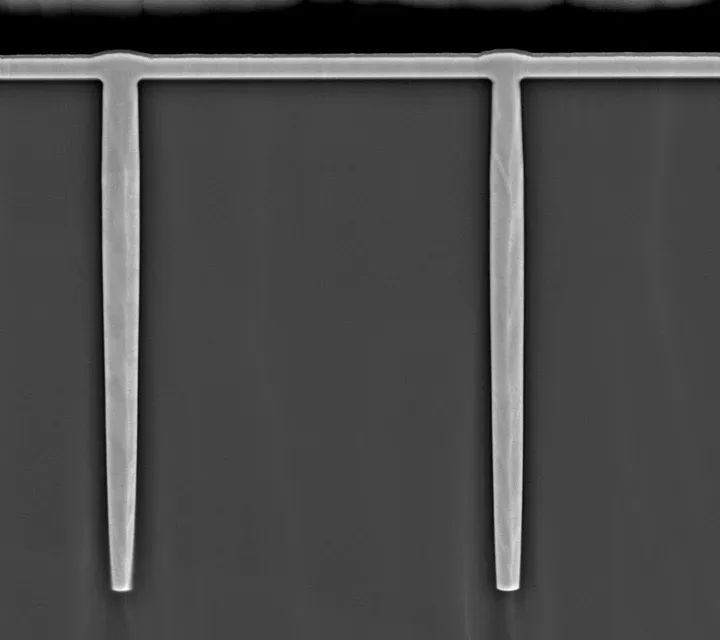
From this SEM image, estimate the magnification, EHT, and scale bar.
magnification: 0.7 K X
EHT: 20 kV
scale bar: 20000 nm